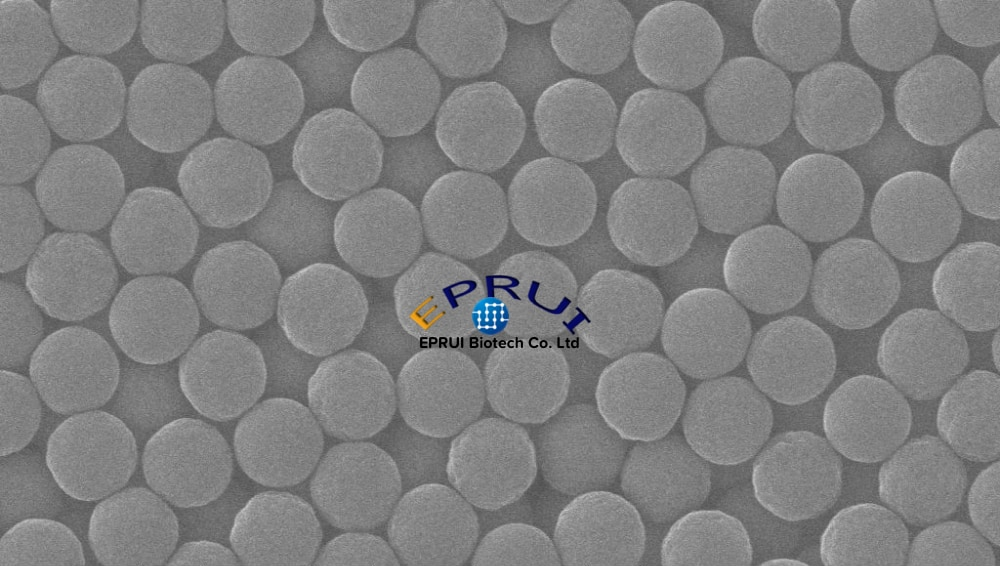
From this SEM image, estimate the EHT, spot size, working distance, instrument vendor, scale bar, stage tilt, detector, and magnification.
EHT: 20 kV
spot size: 3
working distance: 8.6 mm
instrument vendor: FEI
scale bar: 2000 nm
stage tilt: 0°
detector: ETD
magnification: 50 K X